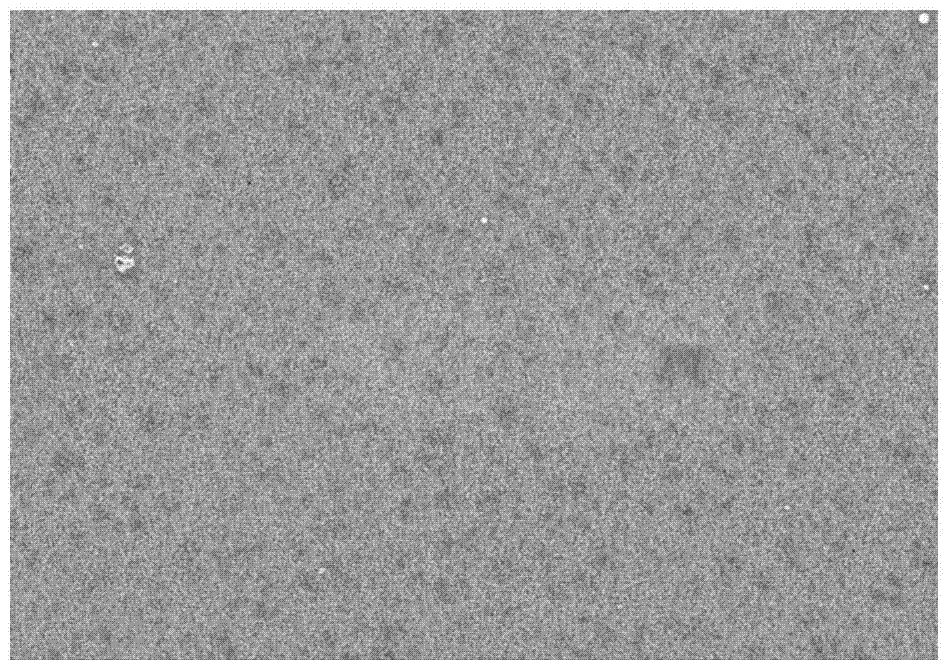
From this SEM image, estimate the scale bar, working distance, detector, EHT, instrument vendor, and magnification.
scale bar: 5000 nm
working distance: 8.6 mm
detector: SE(M)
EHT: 3 kV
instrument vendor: Hitachi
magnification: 10 K X